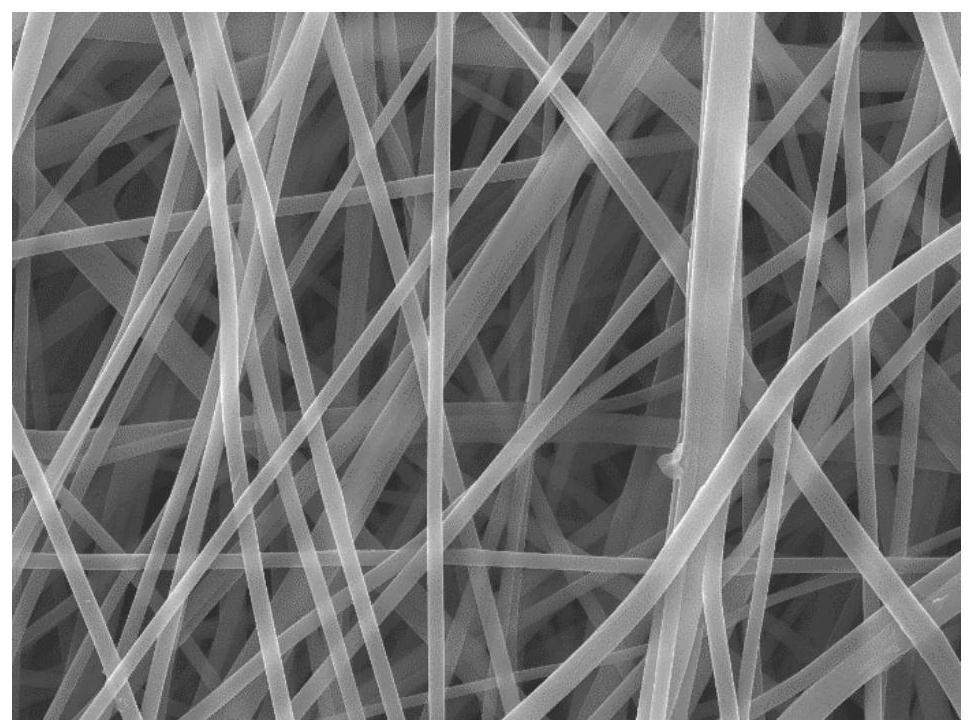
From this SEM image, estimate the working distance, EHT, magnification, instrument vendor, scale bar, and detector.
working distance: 9.6 mm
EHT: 20 kV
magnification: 5 K X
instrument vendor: JEOL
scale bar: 1000 nm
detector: SEI